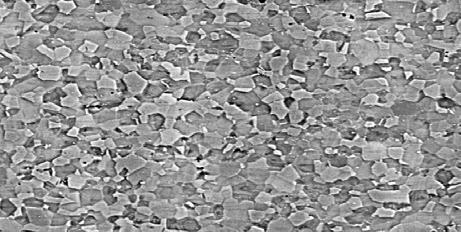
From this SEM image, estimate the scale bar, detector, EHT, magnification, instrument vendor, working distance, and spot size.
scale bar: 50000 nm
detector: BSE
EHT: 30 kV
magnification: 0.5 K X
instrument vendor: FEI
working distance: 10.6 mm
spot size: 5